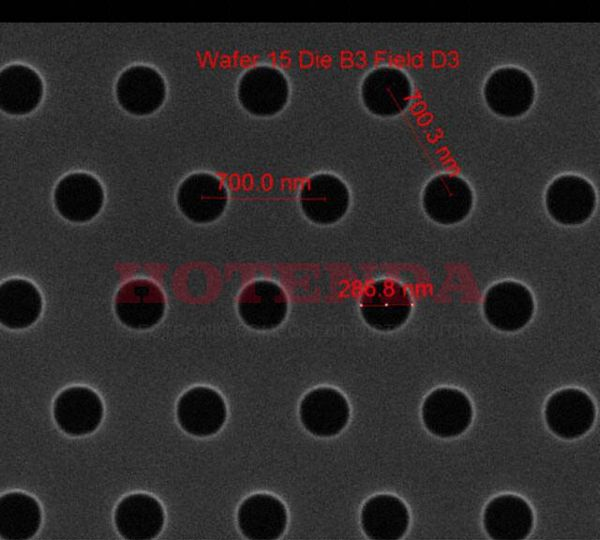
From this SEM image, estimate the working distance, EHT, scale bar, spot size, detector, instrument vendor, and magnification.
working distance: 10.2 mm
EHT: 10 kV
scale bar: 1000 nm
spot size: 1.5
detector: ETD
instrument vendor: FEI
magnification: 75 K X